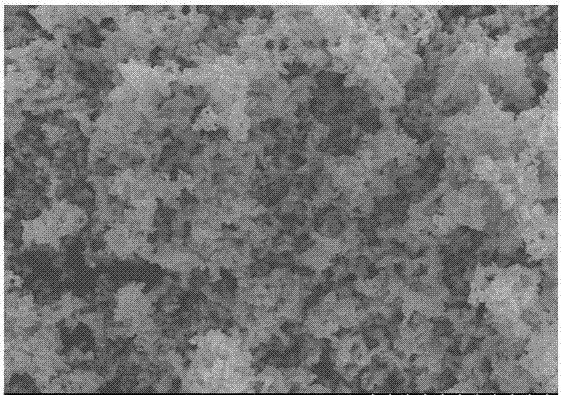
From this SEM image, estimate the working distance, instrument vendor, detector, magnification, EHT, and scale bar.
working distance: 11.4 mm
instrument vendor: Hitachi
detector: SE(M)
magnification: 20 K X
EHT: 5 kV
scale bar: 2000 nm